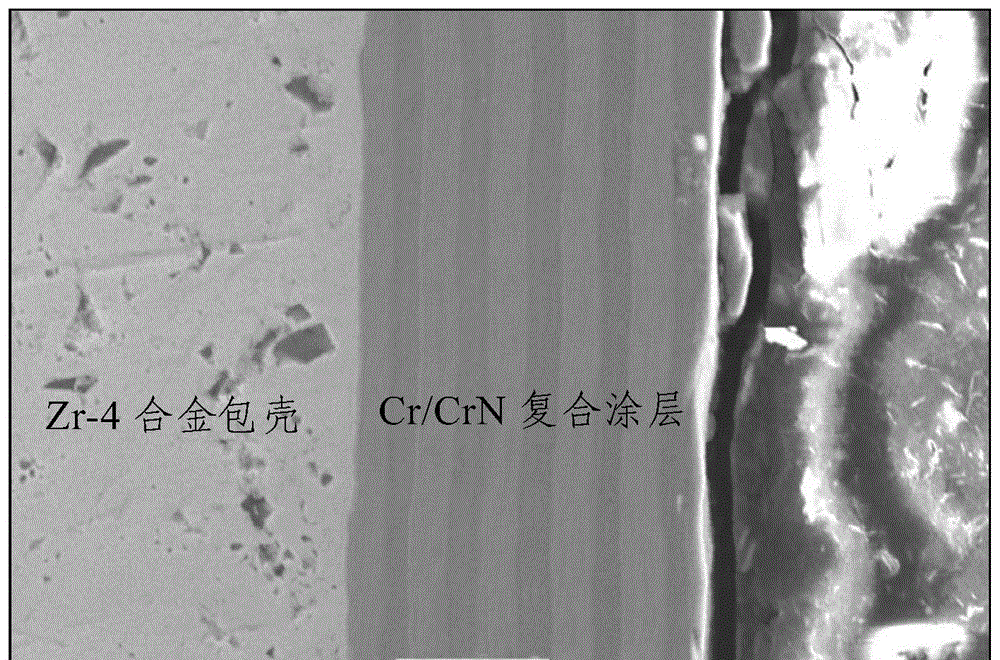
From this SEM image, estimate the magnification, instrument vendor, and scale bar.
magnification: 2 K X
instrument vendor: JEOL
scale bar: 10000 nm